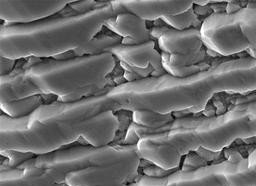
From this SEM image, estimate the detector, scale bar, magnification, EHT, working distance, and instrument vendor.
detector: SED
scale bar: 1000 nm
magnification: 20 K X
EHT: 7 kV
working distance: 8.8 mm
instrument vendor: JEOL